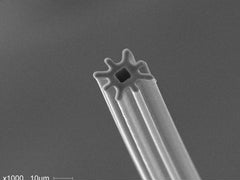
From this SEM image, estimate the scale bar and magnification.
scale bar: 10000 nm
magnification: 1 K X